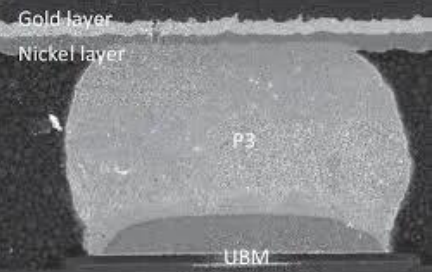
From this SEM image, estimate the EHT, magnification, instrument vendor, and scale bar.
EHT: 10 kV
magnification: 3.3 K X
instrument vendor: JEOL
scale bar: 5000 nm